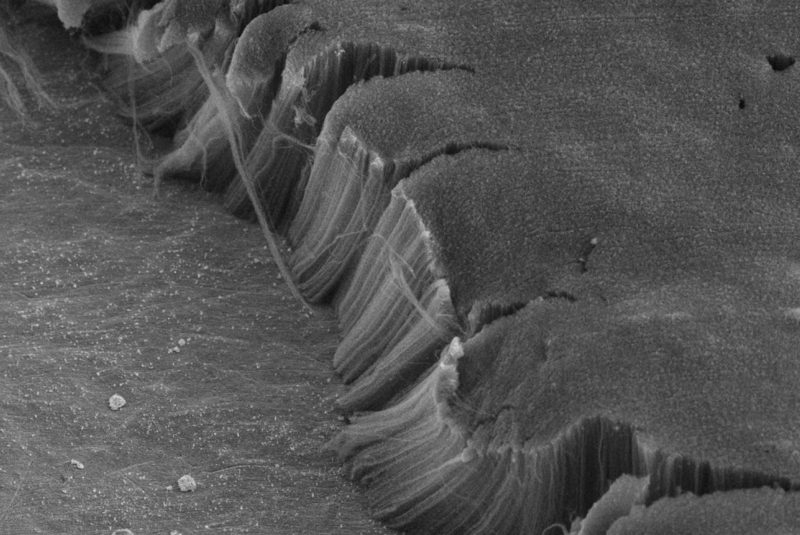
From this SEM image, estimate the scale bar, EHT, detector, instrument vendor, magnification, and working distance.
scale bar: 10000 nm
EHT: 5 kV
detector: SE2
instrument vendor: Zeiss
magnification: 2 K X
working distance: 10 mm